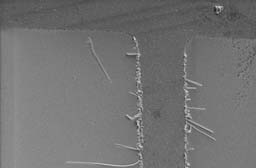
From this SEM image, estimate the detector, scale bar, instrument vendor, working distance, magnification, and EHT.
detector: SE1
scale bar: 10000 nm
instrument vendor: Zeiss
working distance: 8 mm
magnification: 1 K X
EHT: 5.3 kV